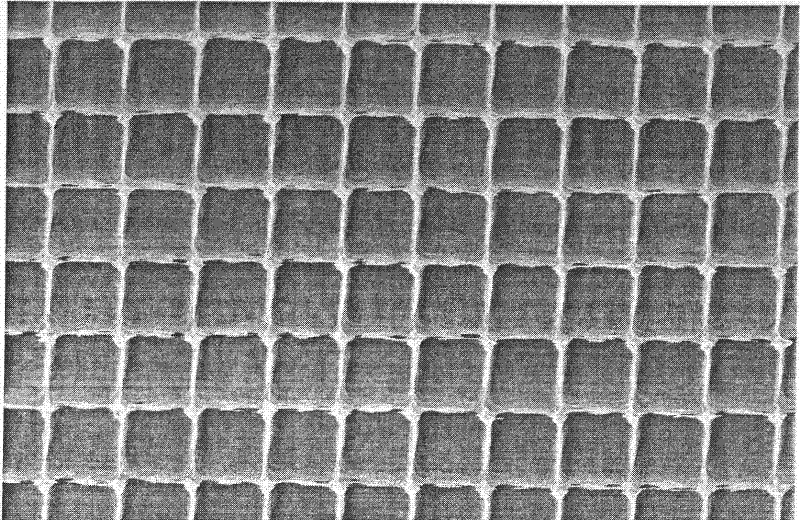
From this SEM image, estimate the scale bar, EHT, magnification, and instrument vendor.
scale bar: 10000 nm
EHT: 10 kV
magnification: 2 K X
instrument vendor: JEOL